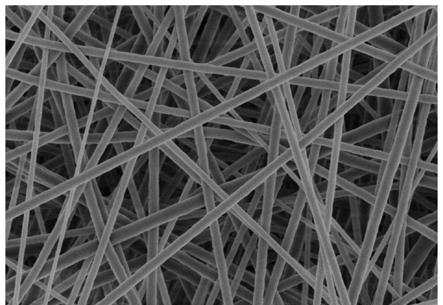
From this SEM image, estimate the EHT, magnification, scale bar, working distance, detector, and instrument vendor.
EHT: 3 kV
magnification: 15 K X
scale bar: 3000 nm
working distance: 7.8 mm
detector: SE(M)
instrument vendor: Hitachi